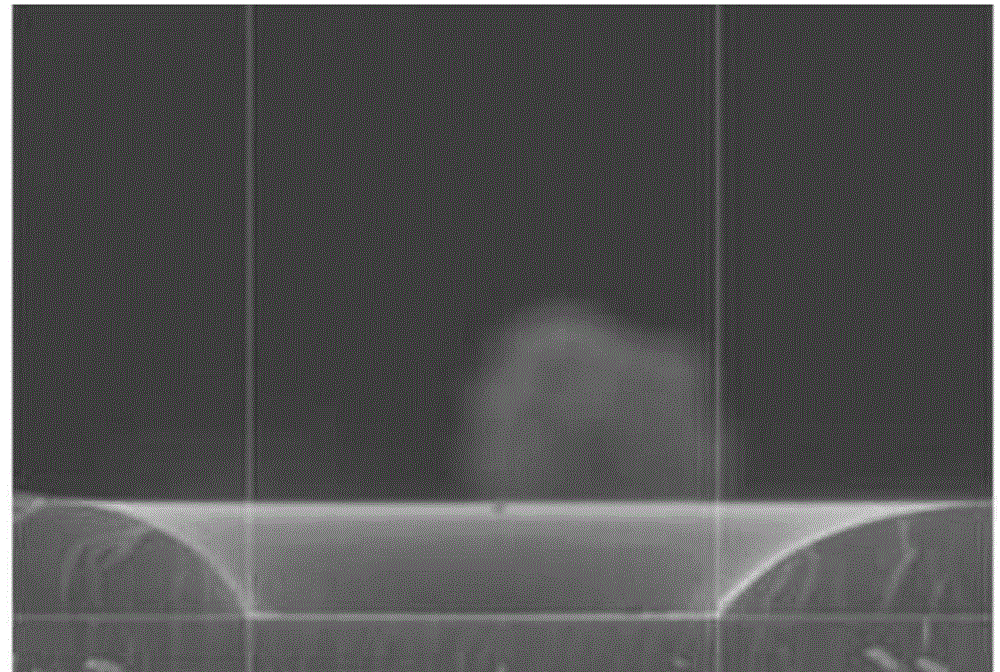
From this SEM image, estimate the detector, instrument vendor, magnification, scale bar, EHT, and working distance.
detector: SEI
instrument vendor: JEOL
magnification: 4 K X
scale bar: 1000 nm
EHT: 15 kV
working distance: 7.3 mm